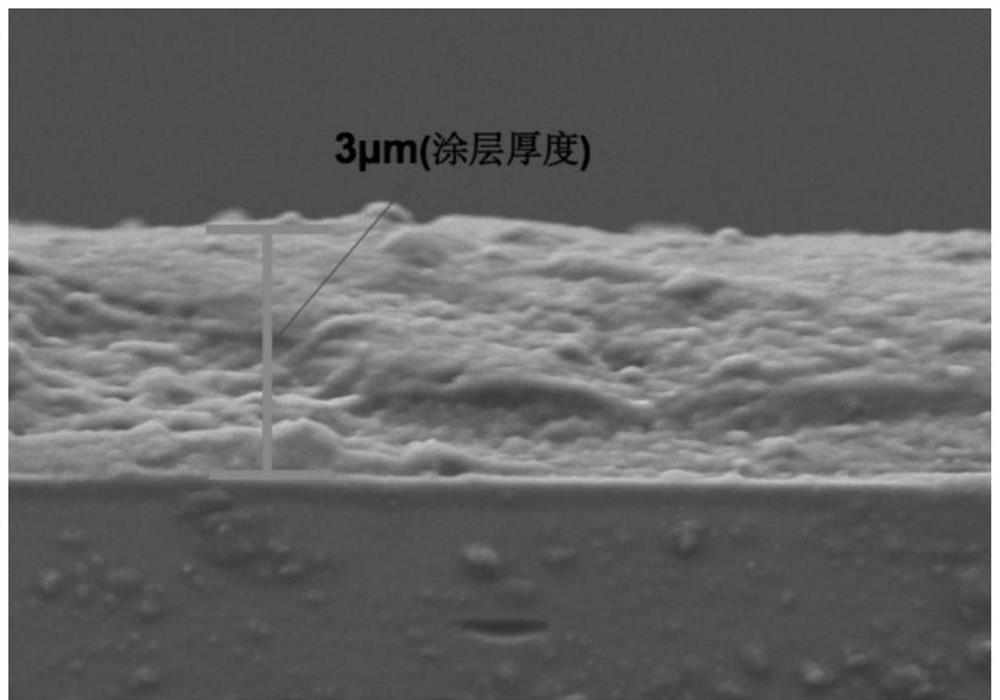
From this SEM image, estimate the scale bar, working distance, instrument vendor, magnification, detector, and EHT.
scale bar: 1000 nm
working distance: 4.1 mm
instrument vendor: Zeiss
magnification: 10 K X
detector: HE-SE2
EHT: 3 kV